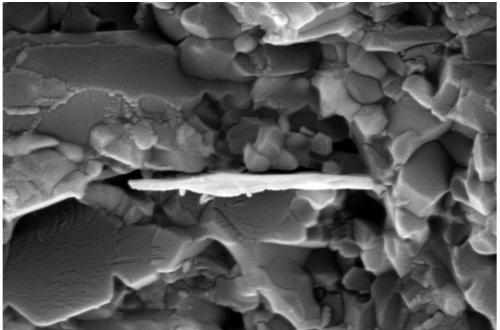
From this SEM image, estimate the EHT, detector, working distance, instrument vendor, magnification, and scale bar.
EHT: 15 kV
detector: SE2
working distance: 9.2 mm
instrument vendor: Zeiss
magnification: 20 K X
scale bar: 200 nm